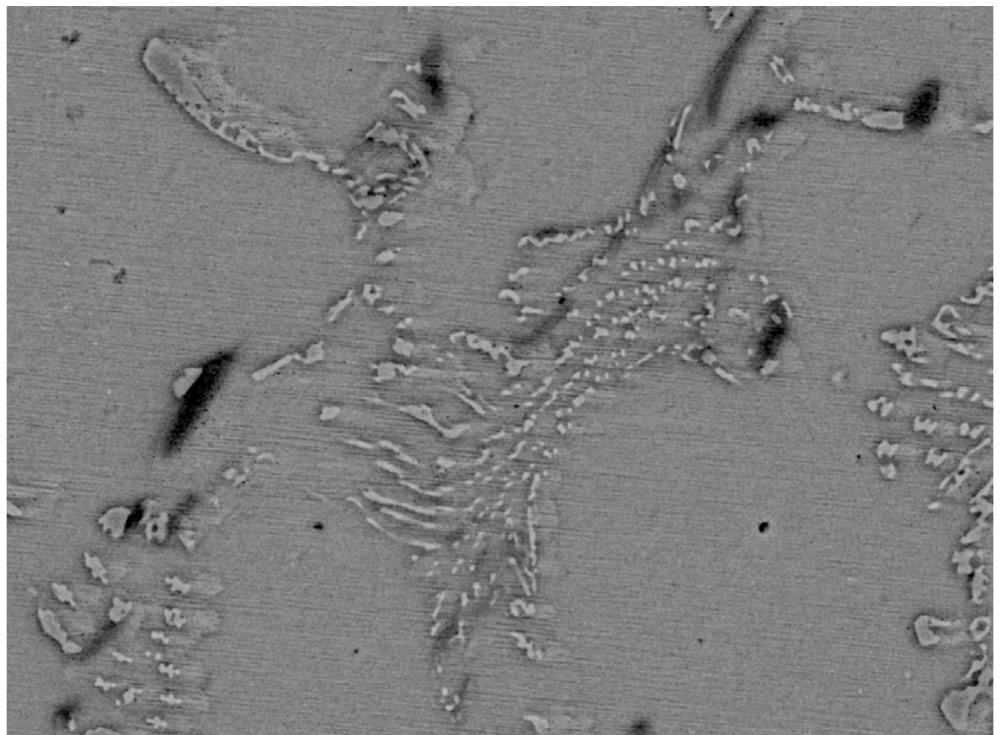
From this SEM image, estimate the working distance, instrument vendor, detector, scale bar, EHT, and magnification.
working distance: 8.8 mm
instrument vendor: JEOL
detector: BED-C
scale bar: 10000 nm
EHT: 20 kV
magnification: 2 K X